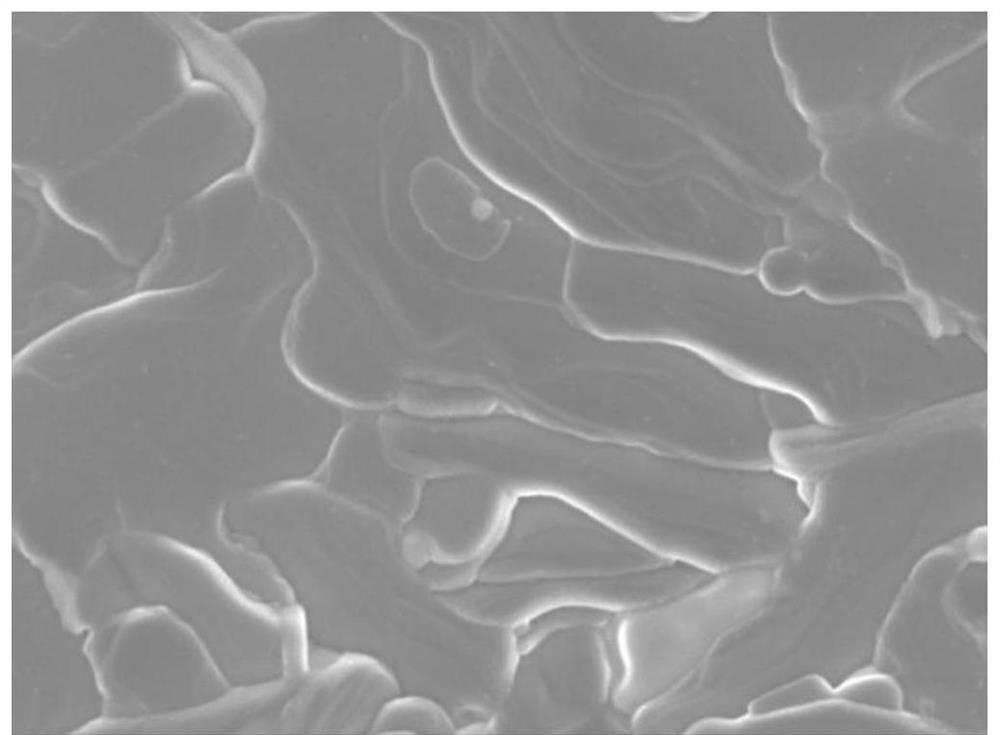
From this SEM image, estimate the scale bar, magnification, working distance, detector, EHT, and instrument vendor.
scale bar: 1000 nm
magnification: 20 K X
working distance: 9.9 mm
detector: SEI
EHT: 3 kV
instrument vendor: JEOL